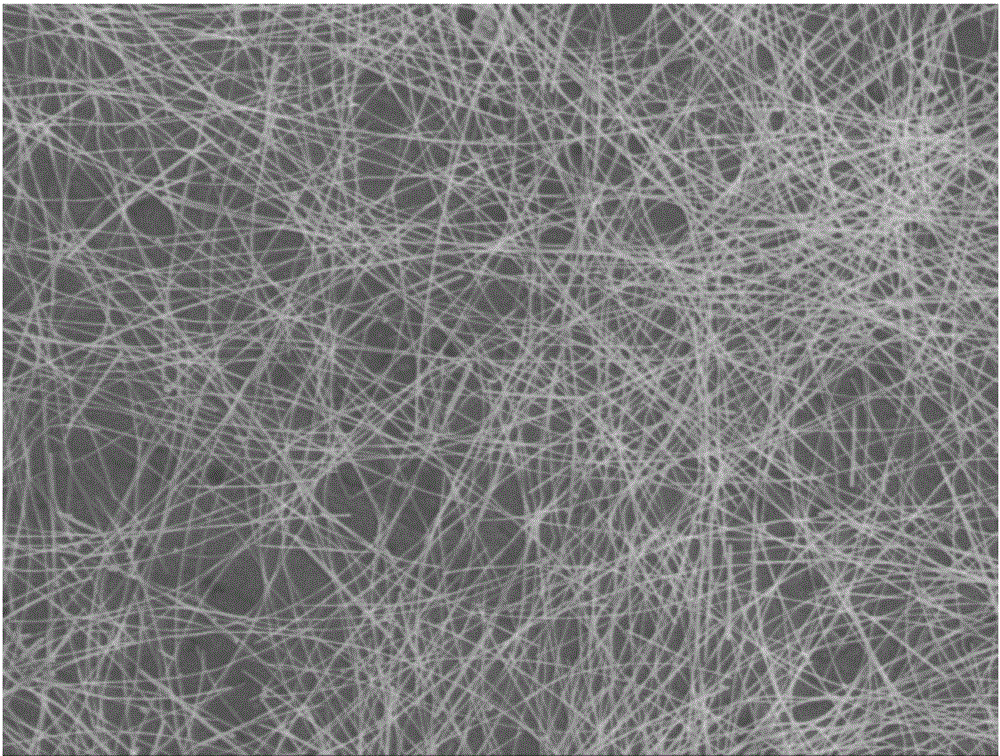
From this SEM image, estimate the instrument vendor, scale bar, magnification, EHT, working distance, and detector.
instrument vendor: JEOL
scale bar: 1000 nm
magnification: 5 K X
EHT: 5 kV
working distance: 8 mm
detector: SEI